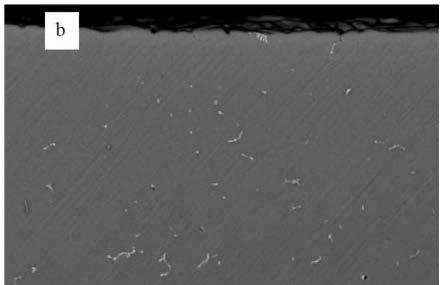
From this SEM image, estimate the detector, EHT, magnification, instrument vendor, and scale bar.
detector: BEC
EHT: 20 kV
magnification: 0.5 K X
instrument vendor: JEOL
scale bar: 50000 nm